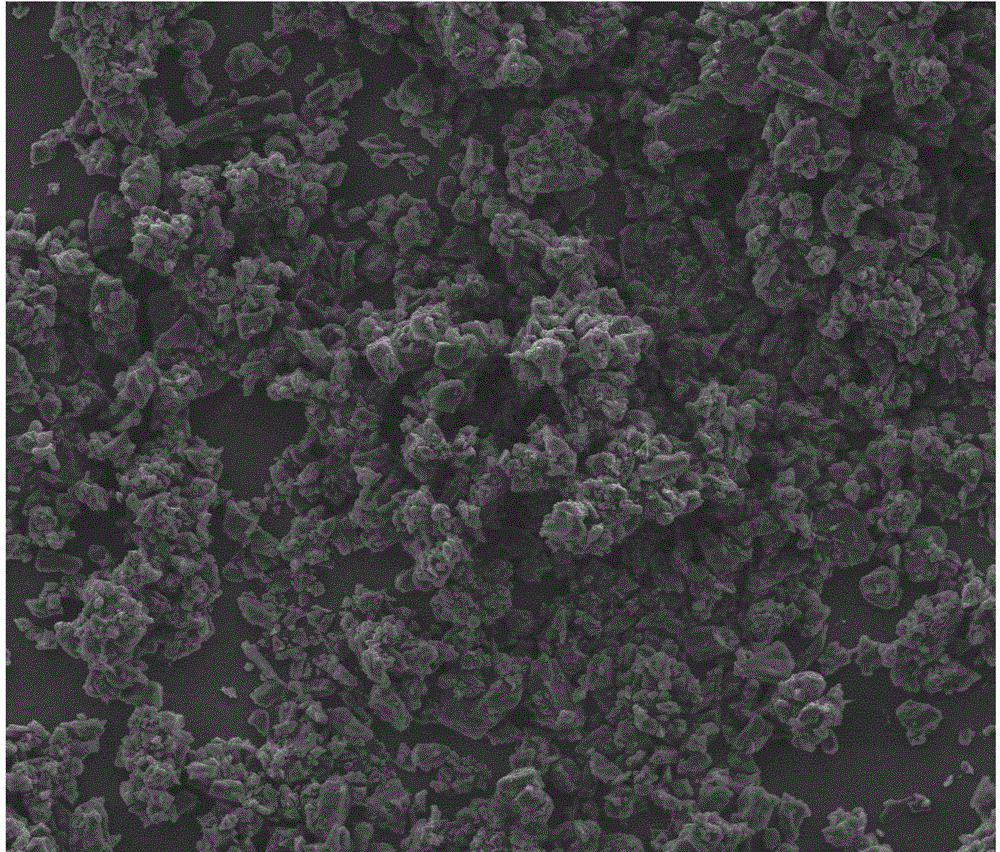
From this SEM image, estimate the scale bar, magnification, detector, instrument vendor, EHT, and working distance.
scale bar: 200000 nm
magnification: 0.5 K X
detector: ETD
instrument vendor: FEI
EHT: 10 kV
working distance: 11.1 mm